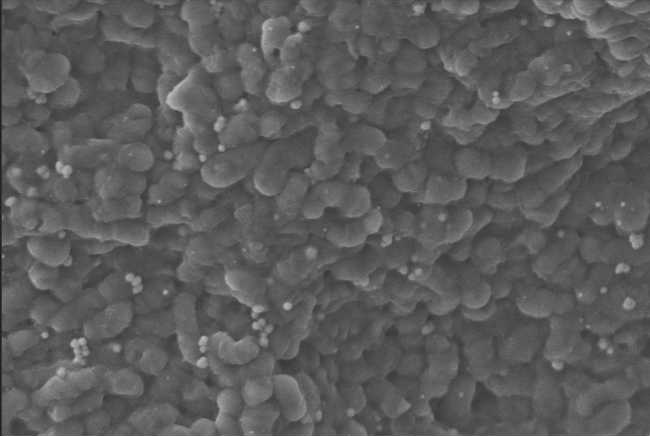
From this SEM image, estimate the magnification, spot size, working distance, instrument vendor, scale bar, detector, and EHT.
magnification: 6.26 K X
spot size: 322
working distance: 6 mm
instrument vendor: Zeiss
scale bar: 1000 nm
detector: SE1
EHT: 5 kV